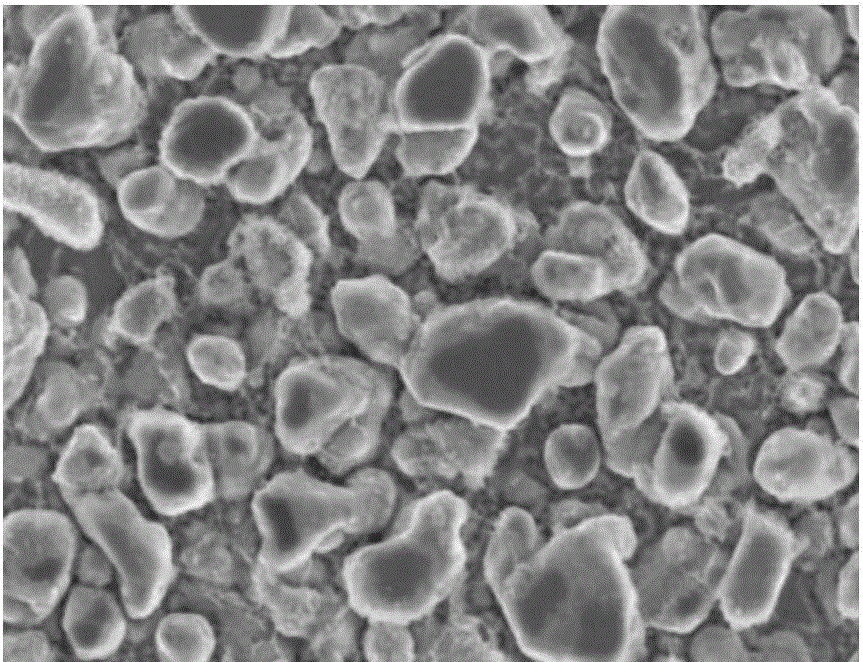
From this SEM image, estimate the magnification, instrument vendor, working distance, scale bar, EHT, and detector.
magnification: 40 K X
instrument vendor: Hitachi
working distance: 10 mm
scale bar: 1000 nm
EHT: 3 kV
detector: SE(M)